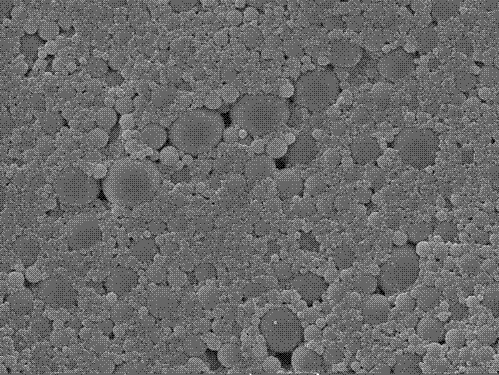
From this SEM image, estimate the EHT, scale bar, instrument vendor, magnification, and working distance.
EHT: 20 kV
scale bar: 20000 nm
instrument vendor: Tescan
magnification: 1 K X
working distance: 12.73 mm